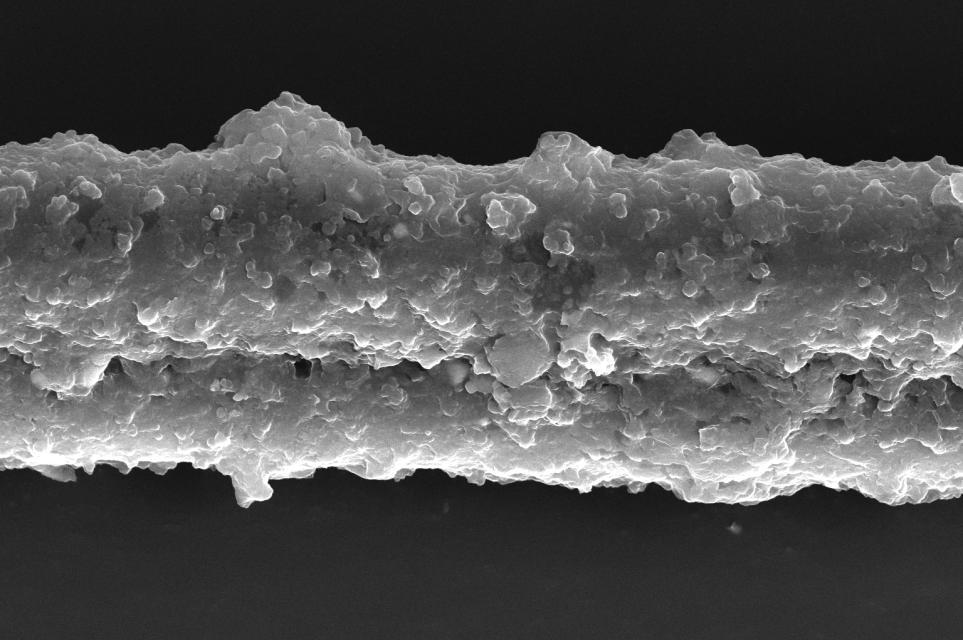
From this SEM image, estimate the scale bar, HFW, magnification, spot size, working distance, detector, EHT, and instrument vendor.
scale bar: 10000 nm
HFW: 41.4 µm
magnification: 10 K X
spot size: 4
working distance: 10.1 mm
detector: ETD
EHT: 20 kV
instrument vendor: FEI